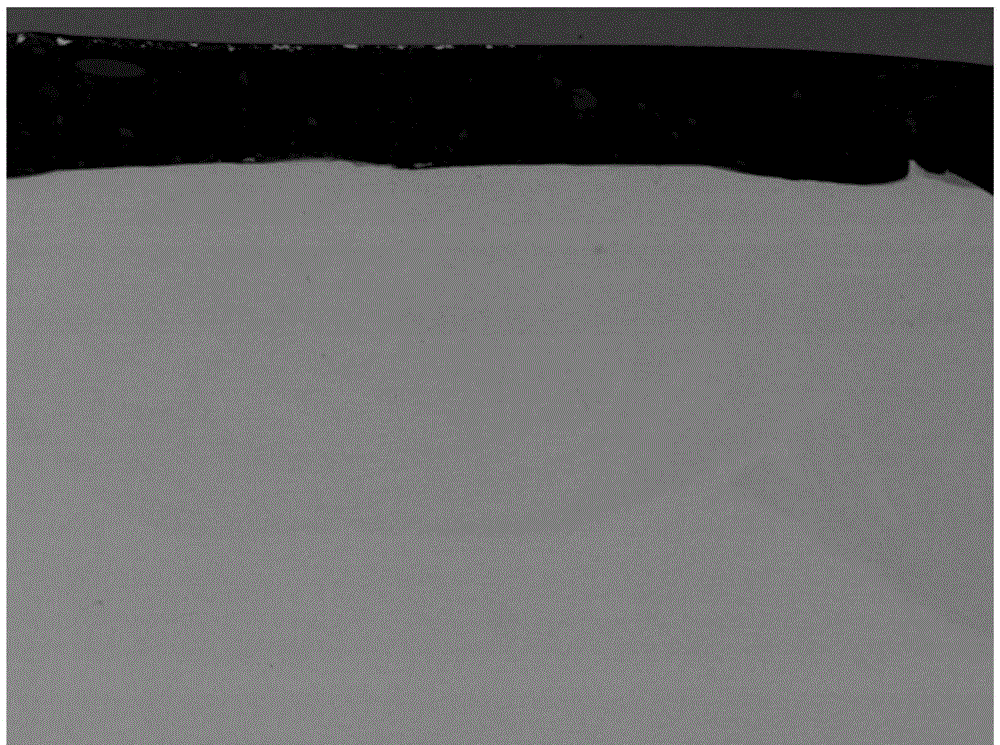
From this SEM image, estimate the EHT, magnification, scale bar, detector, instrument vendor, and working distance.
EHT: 15 kV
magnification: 0.2 K X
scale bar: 100000 nm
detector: COMPO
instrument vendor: JEOL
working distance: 9.9 mm